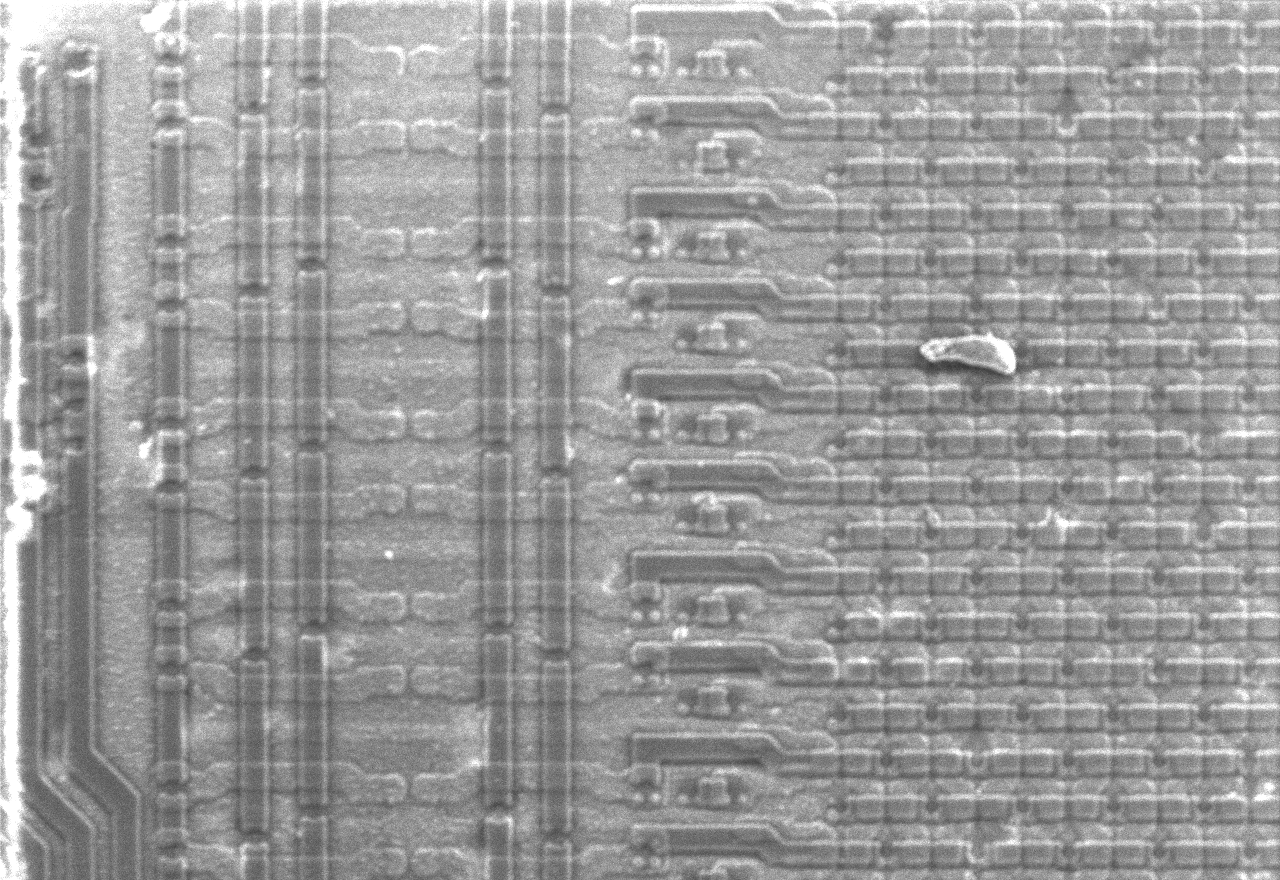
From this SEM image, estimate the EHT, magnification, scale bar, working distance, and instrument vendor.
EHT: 8 kV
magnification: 0.31 K X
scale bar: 32200 nm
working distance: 8.4 mm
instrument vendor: Hitachi